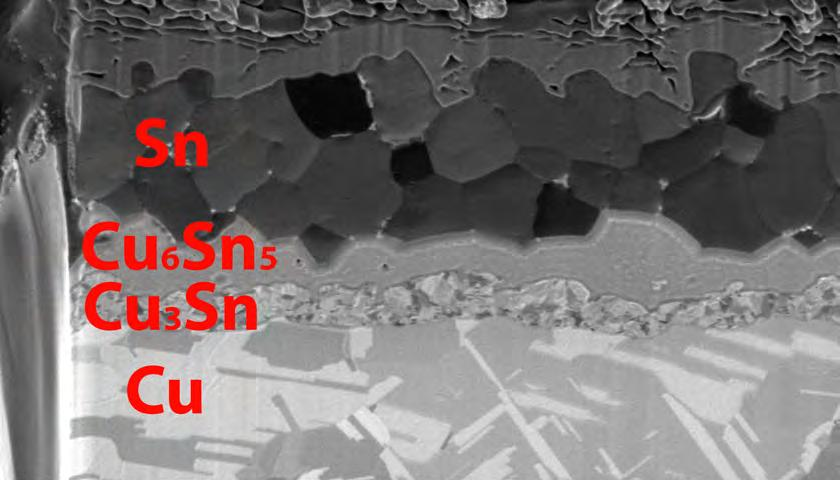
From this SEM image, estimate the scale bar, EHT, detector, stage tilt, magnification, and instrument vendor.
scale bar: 4000 nm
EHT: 30 kV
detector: ETD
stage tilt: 52°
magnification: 12 K X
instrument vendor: FEI